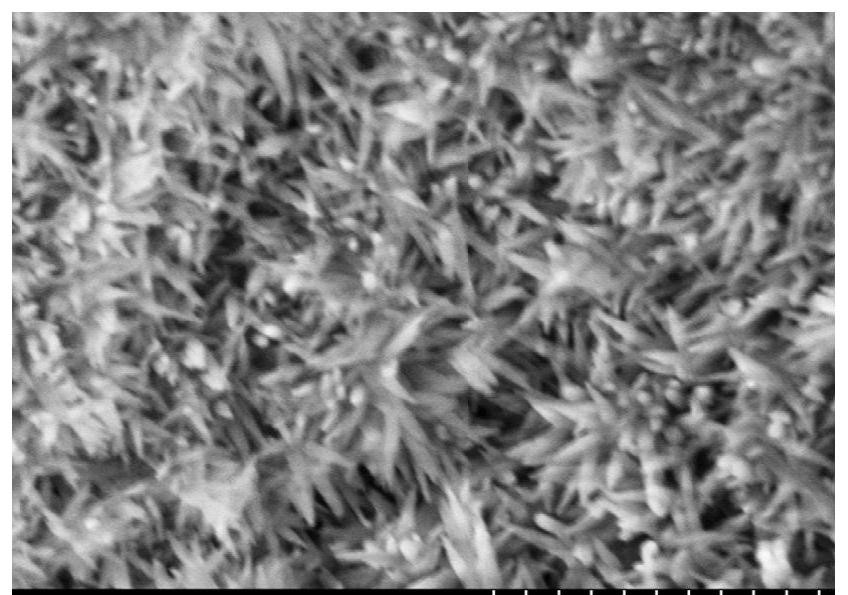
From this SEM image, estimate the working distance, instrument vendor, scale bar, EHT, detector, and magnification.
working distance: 9.5 mm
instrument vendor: Hitachi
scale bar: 1000 nm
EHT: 5 kV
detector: SE(M,LA0)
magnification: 50 K X